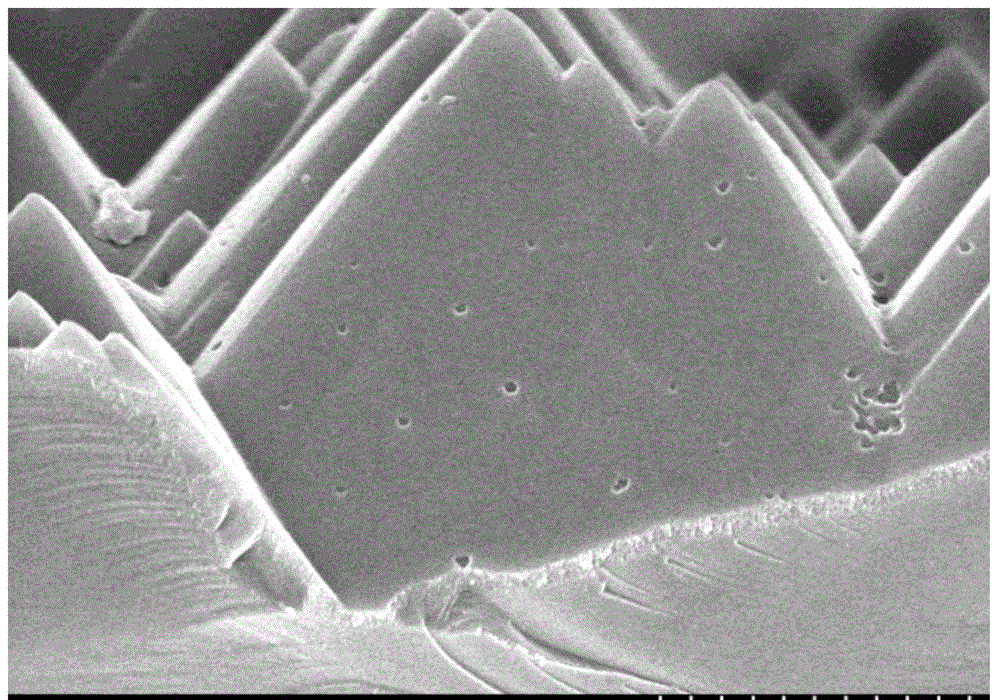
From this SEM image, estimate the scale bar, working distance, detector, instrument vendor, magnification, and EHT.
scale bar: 2000 nm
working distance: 9 mm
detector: SE(M)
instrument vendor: Hitachi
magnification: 20 K X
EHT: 5 kV